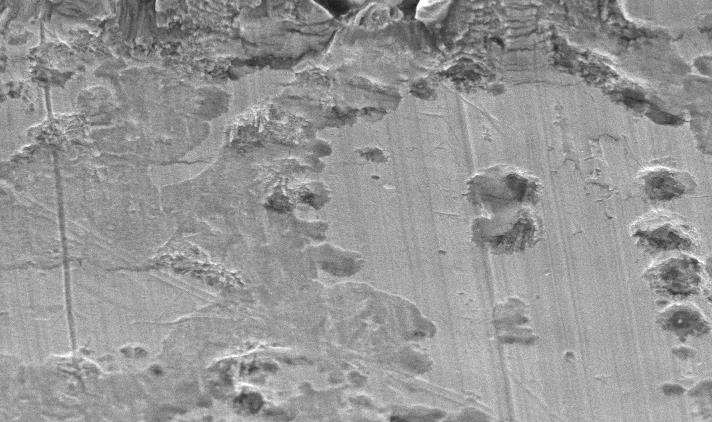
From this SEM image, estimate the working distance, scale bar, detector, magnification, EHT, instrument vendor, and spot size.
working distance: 11.9 mm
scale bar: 20000 nm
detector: SE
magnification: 1 K X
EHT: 20 kV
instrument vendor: FEI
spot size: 4